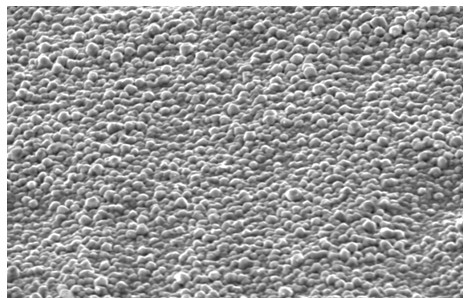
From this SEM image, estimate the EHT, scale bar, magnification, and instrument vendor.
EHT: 20 kV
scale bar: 10000 nm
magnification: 2 K X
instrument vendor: other